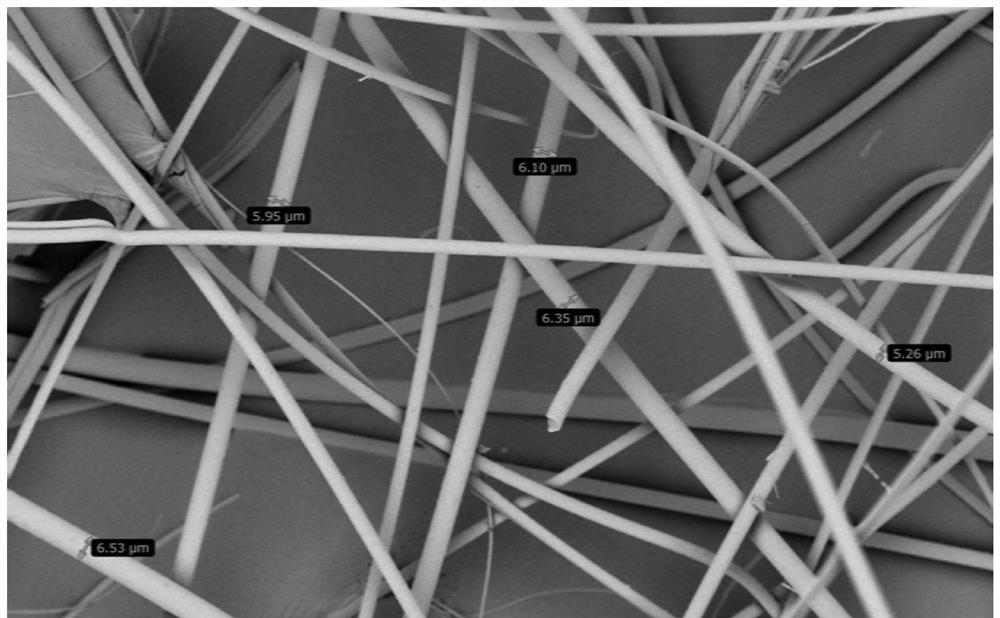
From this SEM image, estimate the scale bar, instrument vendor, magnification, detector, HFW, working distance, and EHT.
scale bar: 80000 nm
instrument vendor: other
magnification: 2 K X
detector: BSD Full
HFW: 259 µm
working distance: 7.312 mm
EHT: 5 kV